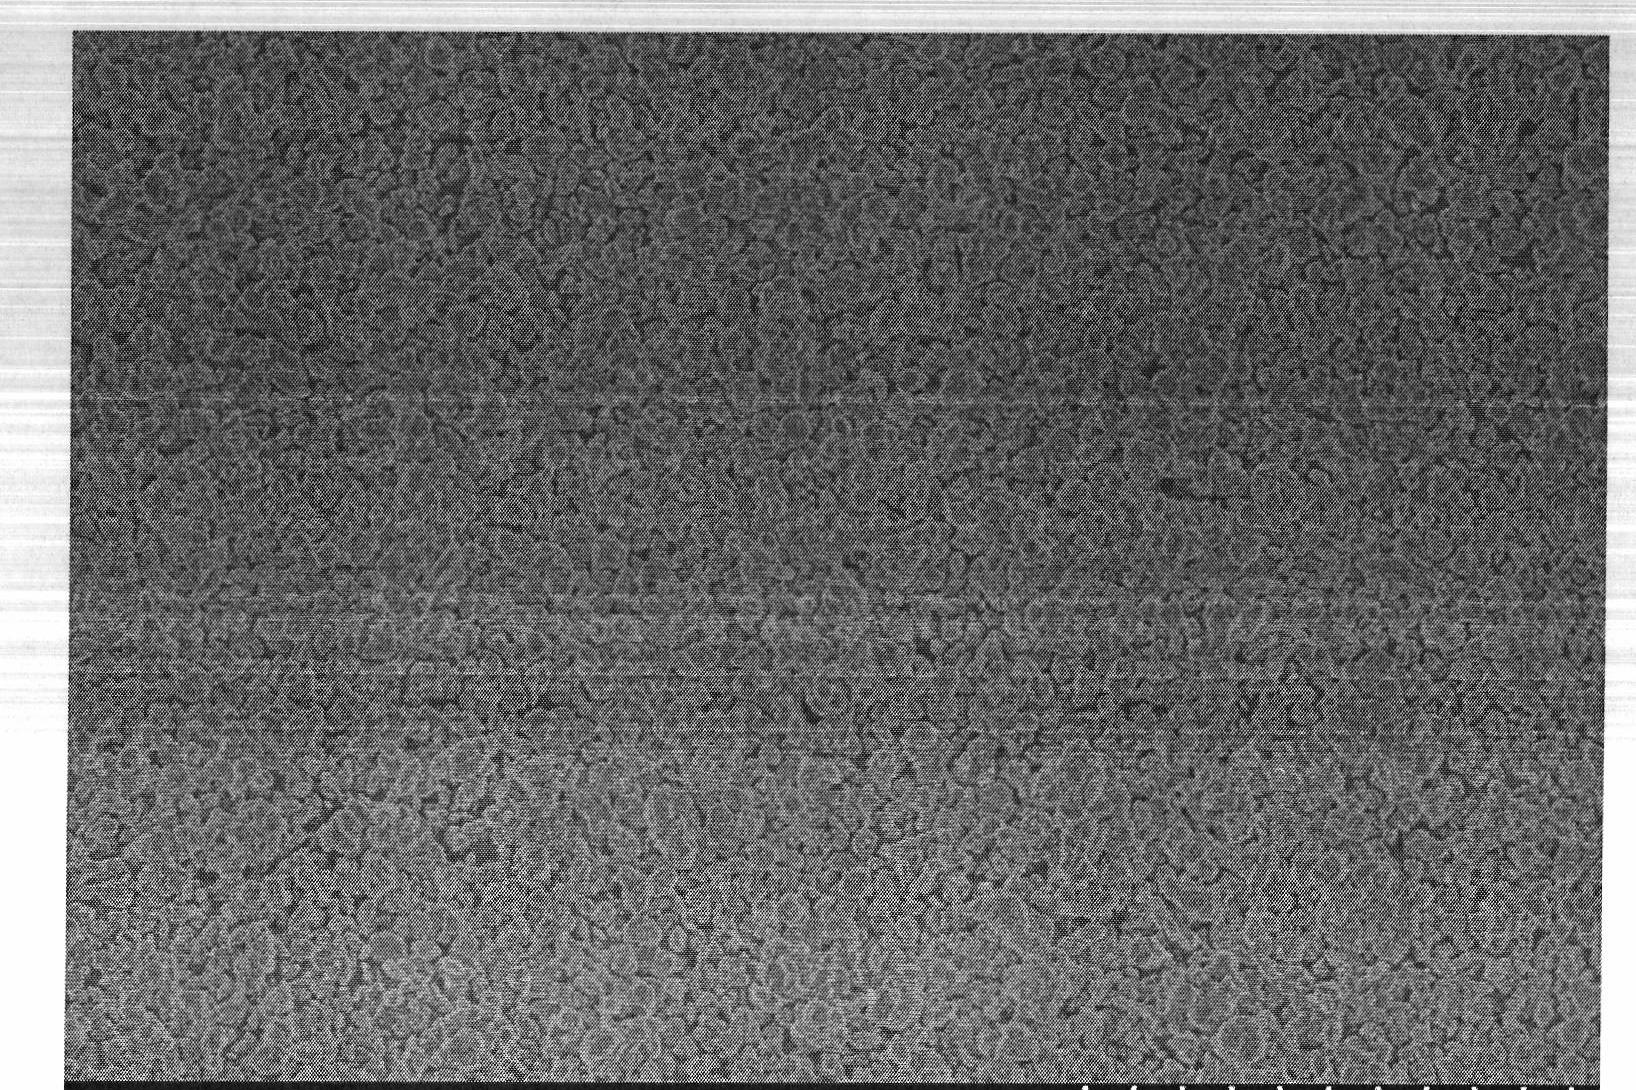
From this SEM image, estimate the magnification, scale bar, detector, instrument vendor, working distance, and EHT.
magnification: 2 K X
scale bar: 20000 nm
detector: SE(U)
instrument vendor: Hitachi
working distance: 4.7 mm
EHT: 4 kV